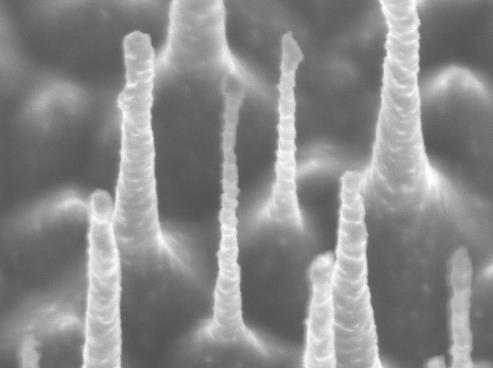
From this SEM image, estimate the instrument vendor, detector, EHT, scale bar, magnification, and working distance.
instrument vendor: JEOL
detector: SEI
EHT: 2 kV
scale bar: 100 nm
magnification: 85 K X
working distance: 3 mm